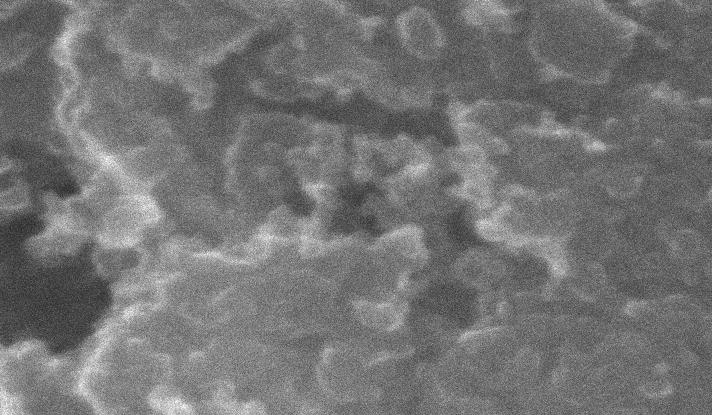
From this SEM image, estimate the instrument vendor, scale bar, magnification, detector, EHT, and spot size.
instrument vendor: FEI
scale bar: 500 nm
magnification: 50 K X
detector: SE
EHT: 20 kV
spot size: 1